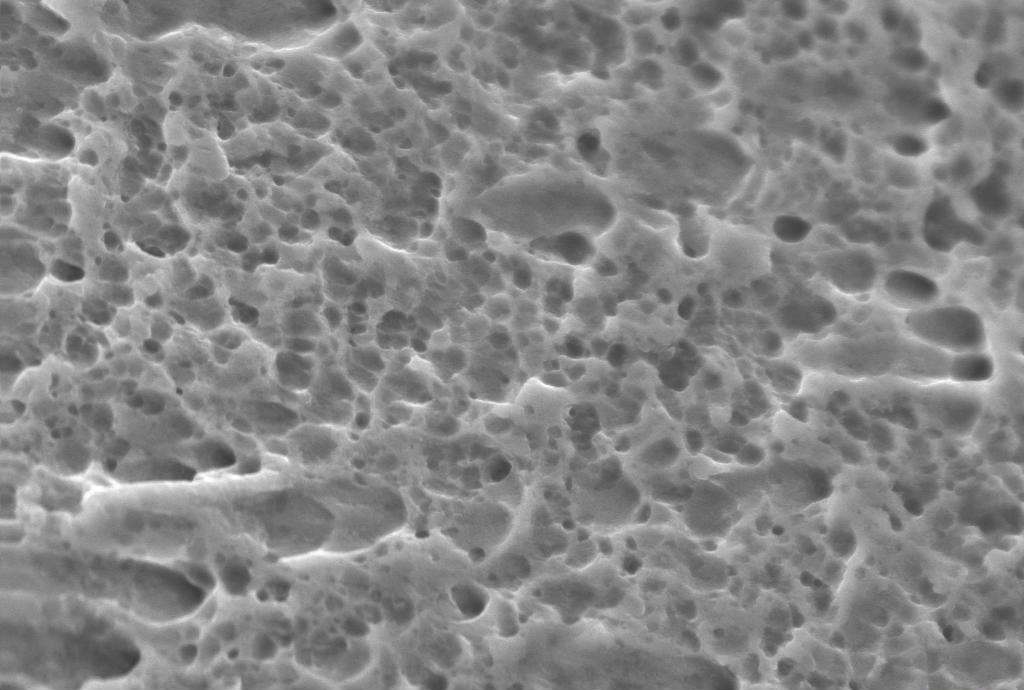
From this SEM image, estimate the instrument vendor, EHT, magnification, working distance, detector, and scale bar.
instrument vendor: Zeiss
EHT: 20 kV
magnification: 4 K X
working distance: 6 mm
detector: SE1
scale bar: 2000 nm